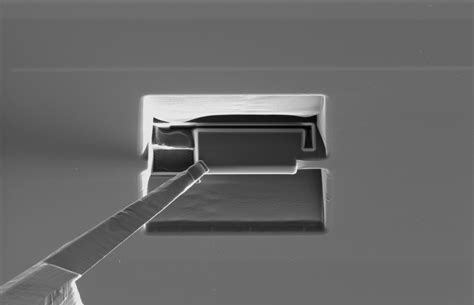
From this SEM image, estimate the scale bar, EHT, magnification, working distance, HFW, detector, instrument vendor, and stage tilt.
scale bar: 10000 nm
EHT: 30 kV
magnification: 2.5 K X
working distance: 11 mm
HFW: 50.8 µm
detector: ETD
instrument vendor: FEI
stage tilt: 0°